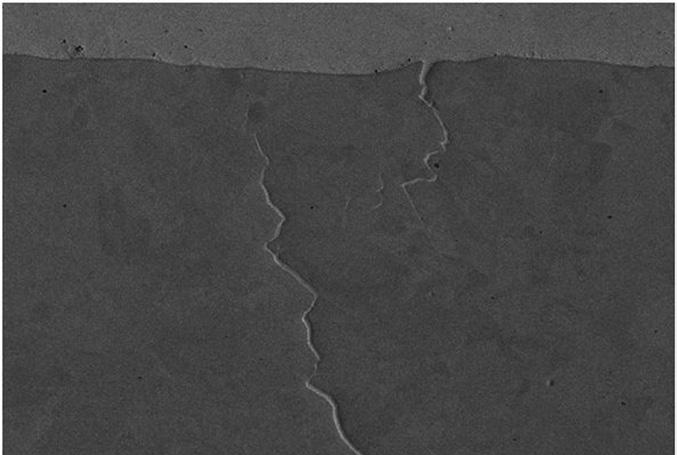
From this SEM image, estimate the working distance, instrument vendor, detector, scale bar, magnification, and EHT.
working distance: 14 mm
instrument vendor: Zeiss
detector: SE2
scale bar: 10000 nm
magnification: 1 K X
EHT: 20 kV